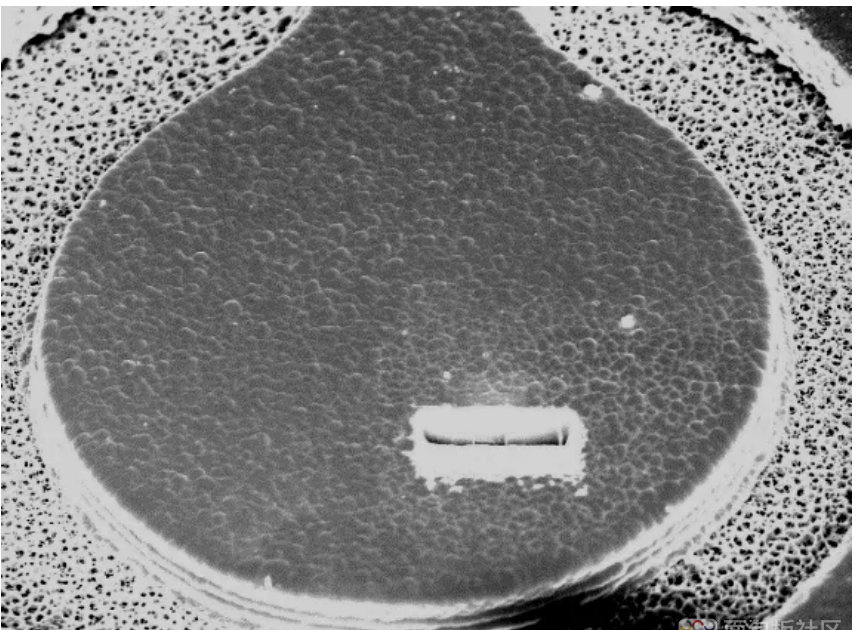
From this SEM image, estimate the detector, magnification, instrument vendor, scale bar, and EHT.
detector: SEI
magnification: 0.43 K X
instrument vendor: JEOL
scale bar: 10000 nm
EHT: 15 kV